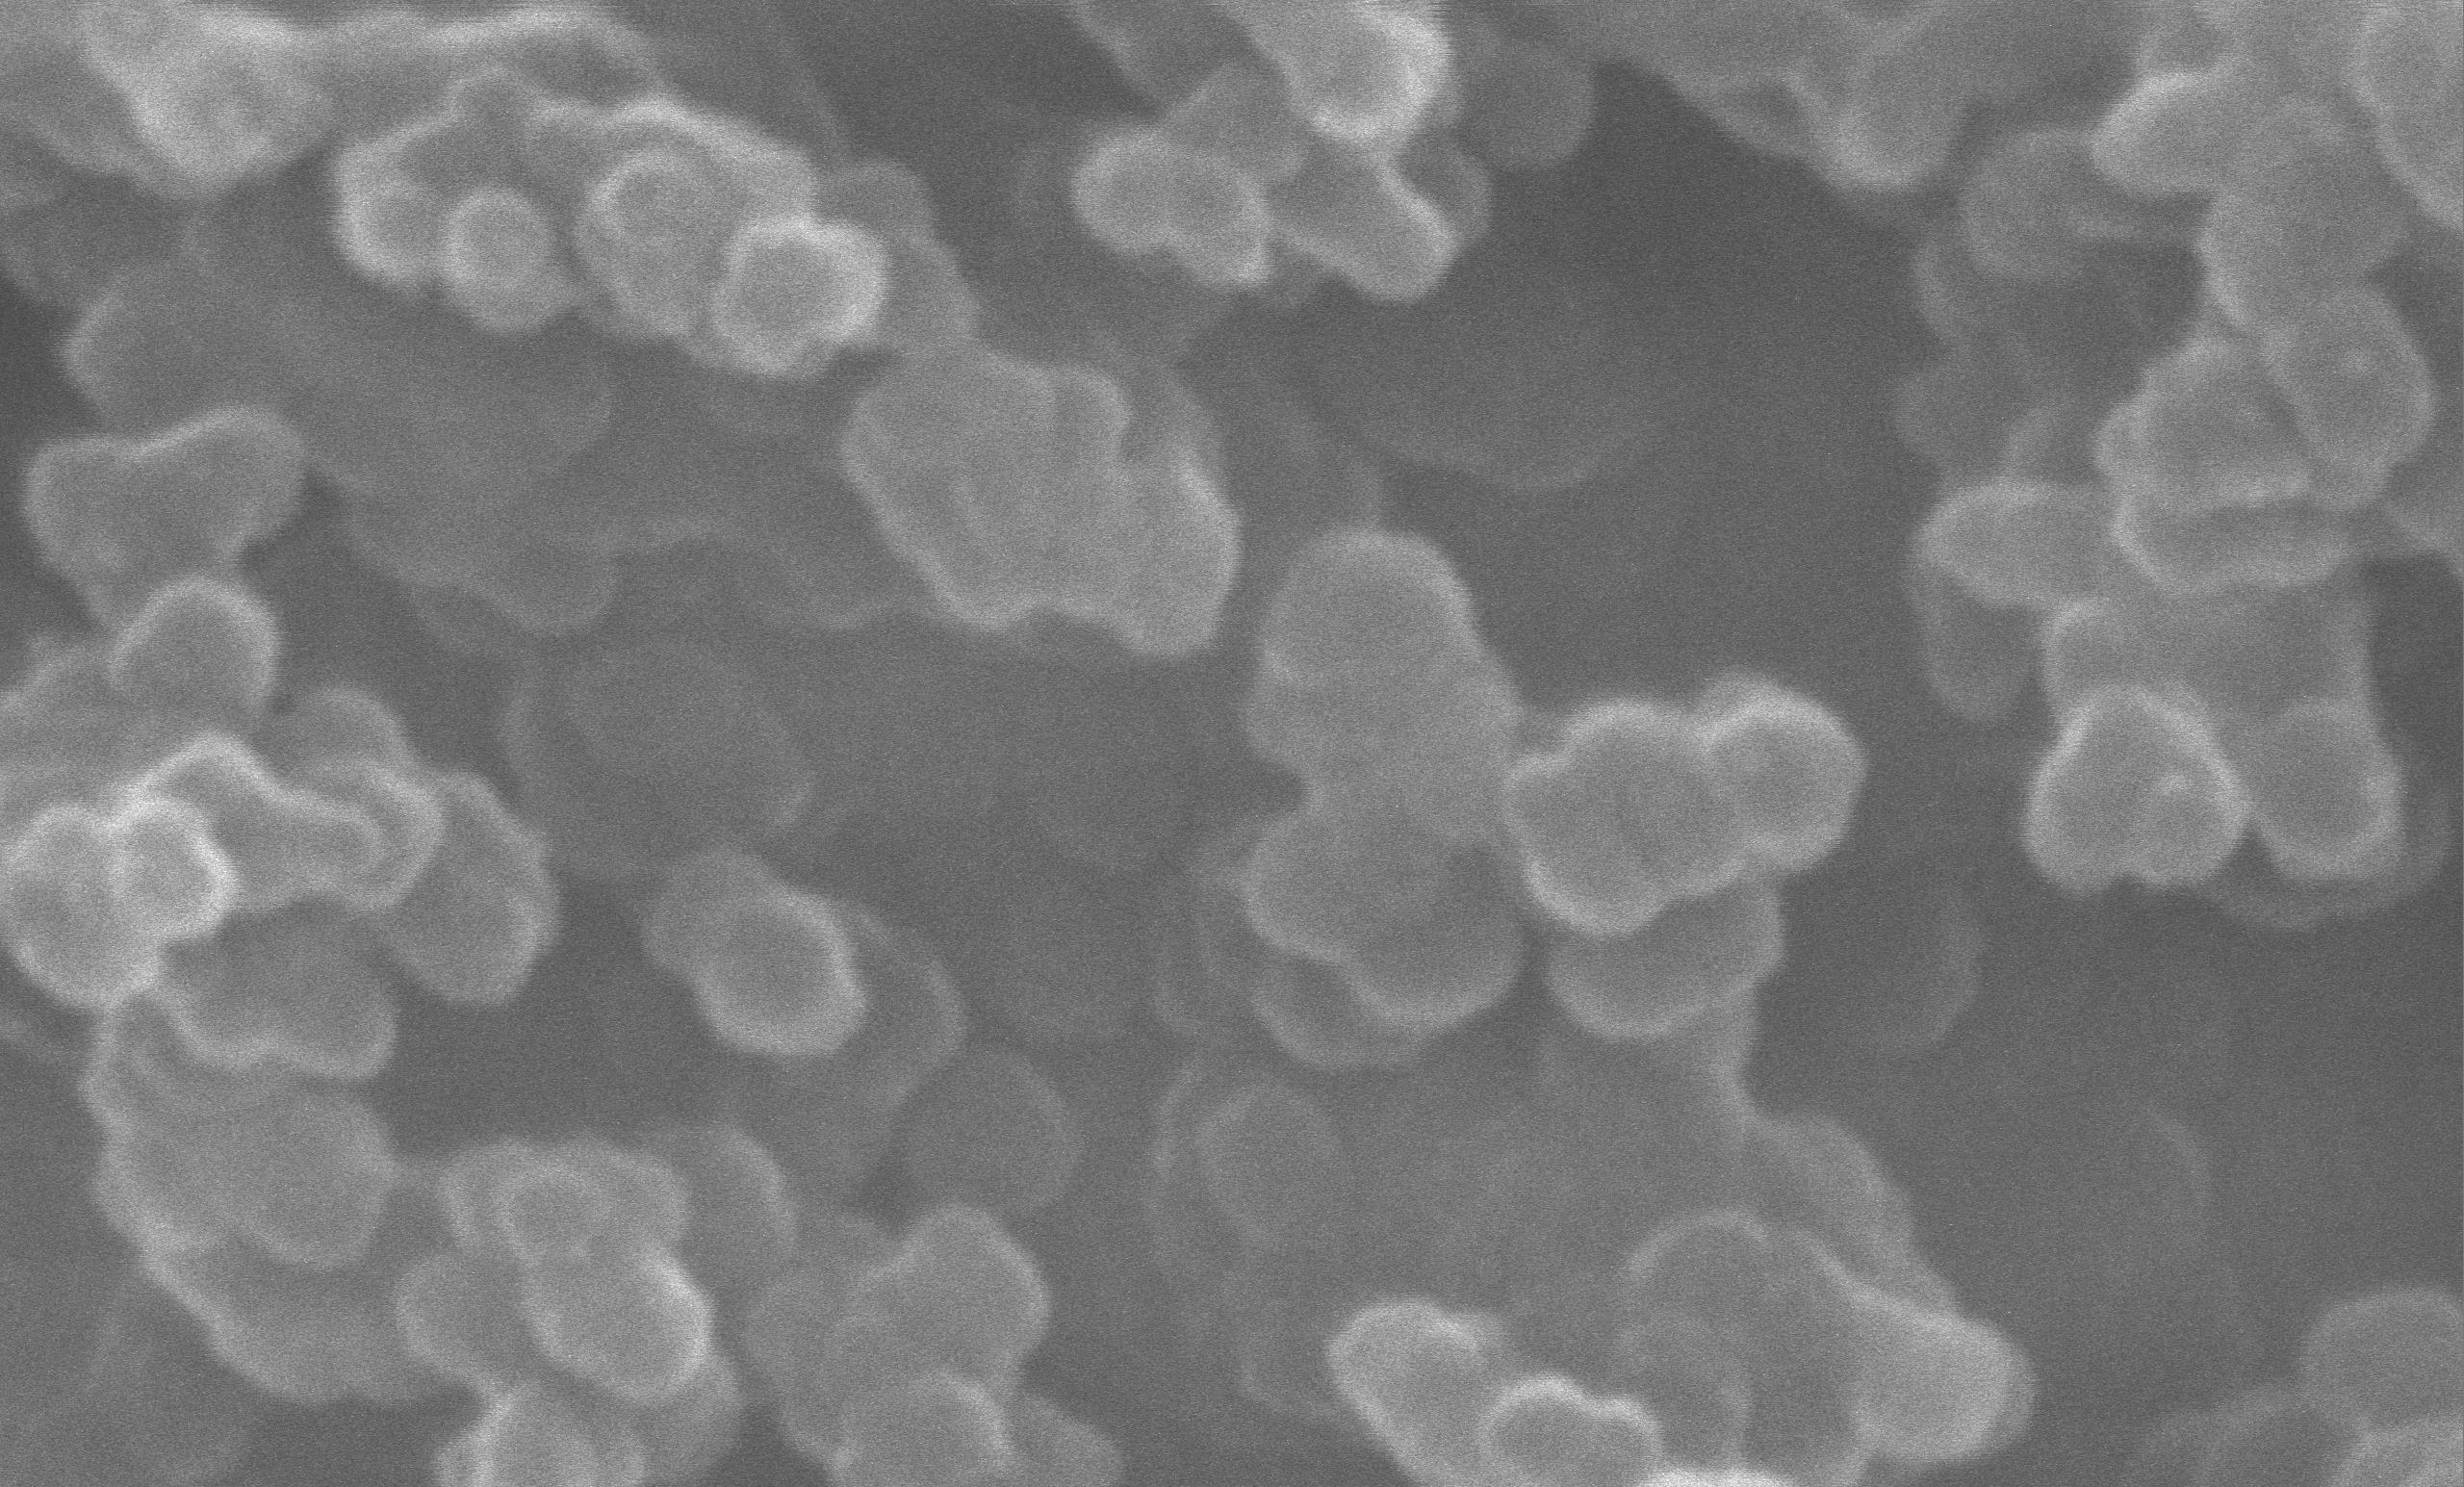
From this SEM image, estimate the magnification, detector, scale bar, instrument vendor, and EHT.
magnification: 50 K X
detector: SE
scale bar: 1000 nm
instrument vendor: JEOL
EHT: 30 kV